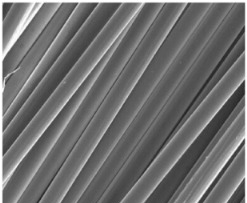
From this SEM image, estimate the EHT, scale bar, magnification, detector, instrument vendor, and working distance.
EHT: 10 kV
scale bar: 100000 nm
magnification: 1 K X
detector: ETD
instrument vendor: FEI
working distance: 9.9 mm